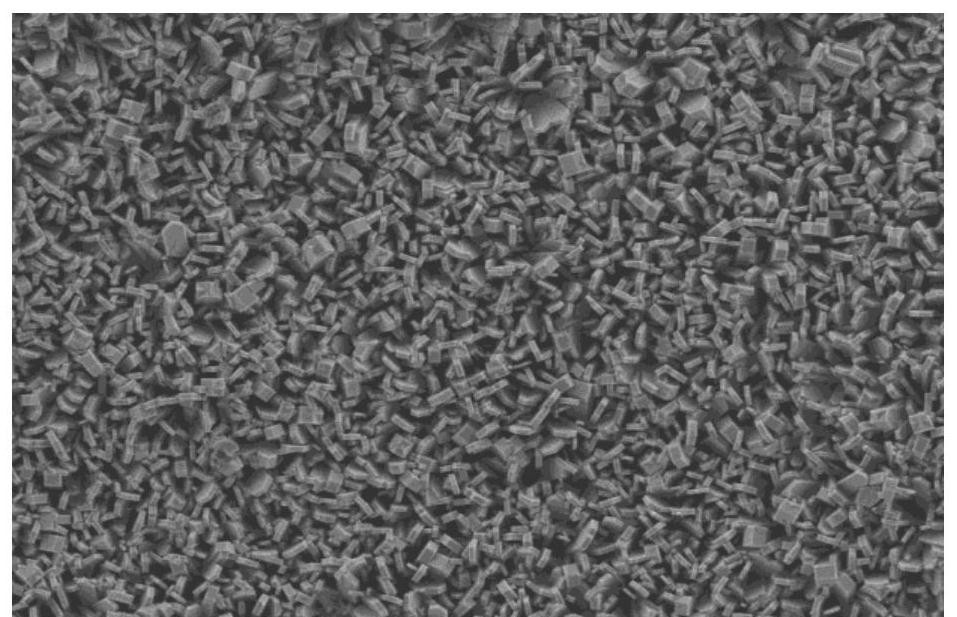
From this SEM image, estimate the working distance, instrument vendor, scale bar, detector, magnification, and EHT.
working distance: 5.2 mm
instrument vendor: Zeiss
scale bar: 3000 nm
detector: SE2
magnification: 2 K X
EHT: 3 kV